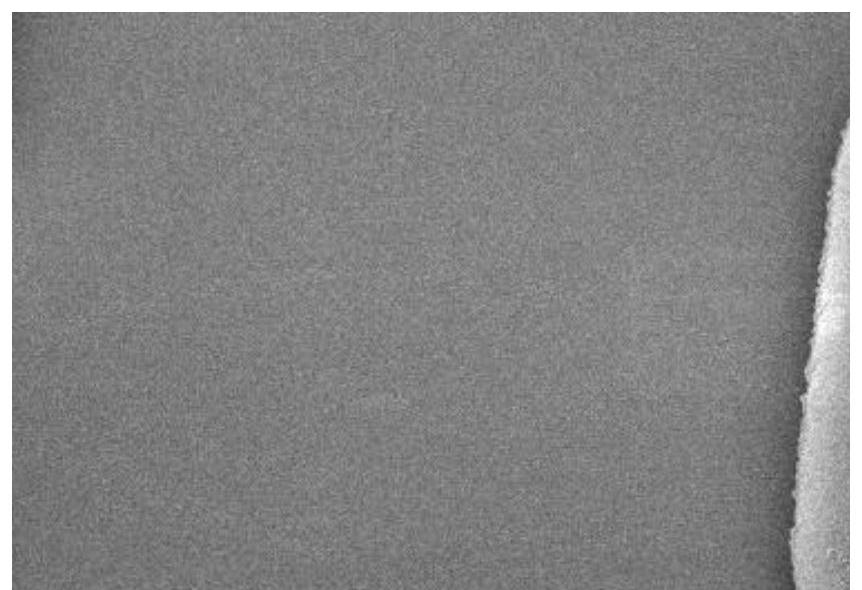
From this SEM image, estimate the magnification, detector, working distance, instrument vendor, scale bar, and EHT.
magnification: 20 K X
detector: SE(UL)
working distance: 14 mm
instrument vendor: Hitachi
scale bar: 2000 nm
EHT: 5 kV